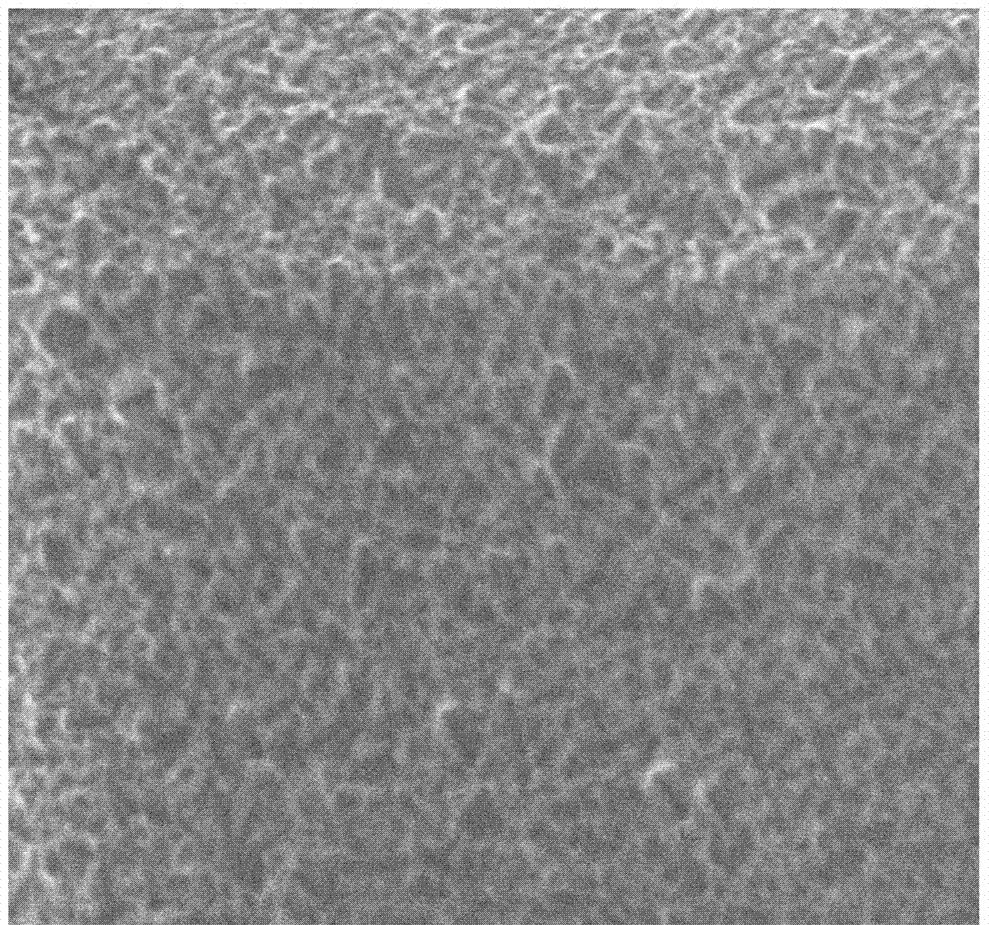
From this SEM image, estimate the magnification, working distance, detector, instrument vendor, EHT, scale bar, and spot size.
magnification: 40 K X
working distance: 7 mm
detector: ETD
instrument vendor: FEI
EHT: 5 kV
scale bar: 3000 nm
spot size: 3